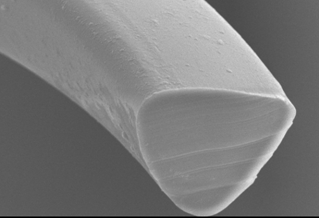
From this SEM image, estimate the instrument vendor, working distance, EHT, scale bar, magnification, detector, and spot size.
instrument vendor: JEOL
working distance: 11 mm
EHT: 10 kV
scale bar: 5000 nm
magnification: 3 K X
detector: SEI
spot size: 50